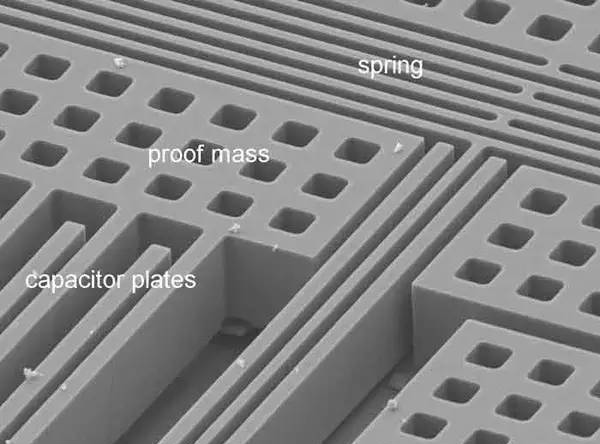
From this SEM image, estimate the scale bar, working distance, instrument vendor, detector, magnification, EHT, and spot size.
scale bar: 20000 nm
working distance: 14.6 mm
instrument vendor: FEI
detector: SE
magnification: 1.5 K X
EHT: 10 kV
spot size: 3